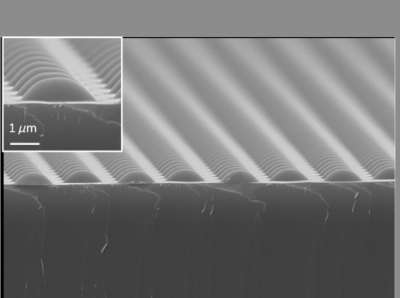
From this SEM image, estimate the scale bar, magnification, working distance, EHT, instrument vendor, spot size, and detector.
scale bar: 5000 nm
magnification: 5 K X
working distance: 11 mm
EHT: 20 kV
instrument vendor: JEOL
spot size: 40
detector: SEI